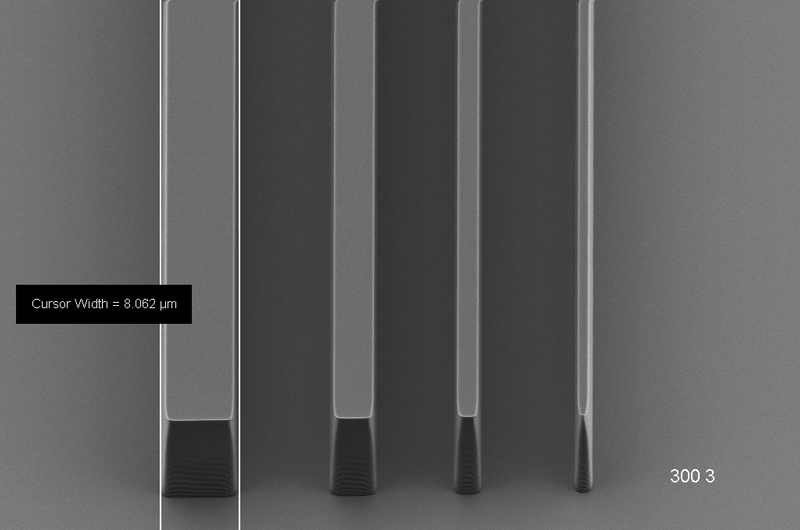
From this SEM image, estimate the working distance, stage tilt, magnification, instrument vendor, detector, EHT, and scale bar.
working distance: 6.7 mm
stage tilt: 45°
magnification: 1.4 K X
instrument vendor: Zeiss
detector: HE-SE2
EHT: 3 kV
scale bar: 10000 nm